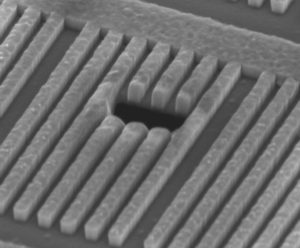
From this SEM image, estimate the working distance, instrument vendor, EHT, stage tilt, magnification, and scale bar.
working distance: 5 mm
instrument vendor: FEI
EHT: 5 kV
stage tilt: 52°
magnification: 56.951 K X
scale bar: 500 nm